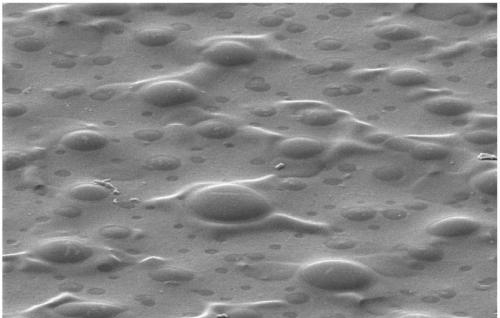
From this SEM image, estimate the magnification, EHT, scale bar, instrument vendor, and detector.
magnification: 5 K X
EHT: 3 kV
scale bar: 10000 nm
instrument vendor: Hitachi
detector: SE(M)